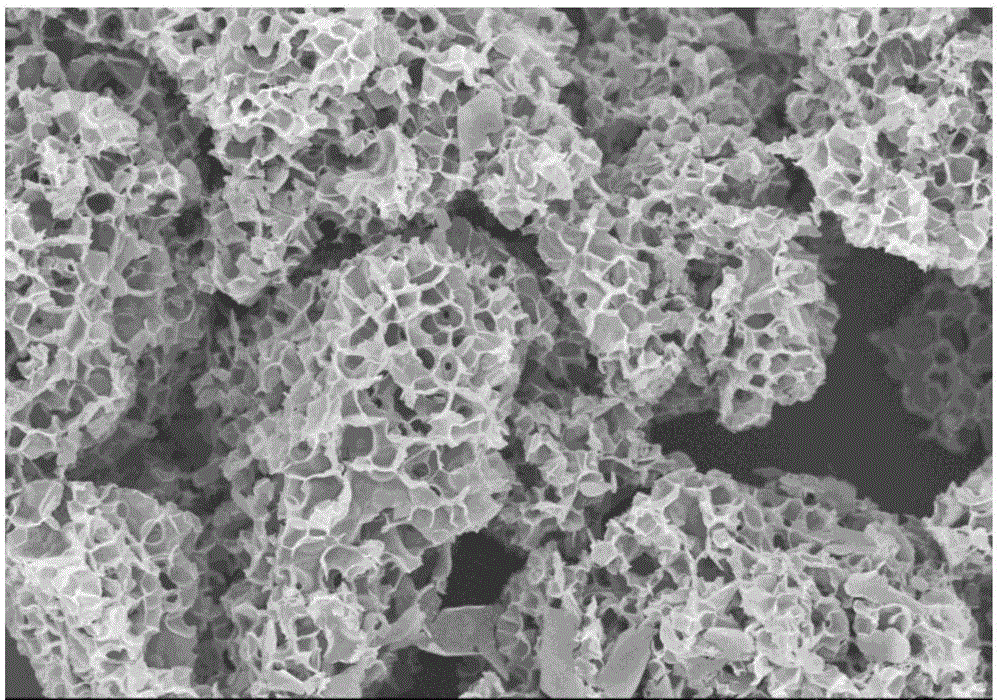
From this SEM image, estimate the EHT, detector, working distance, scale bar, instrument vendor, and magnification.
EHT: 5 kV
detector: SE(U)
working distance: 9.3 mm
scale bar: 20000 nm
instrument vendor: Hitachi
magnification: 2 K X